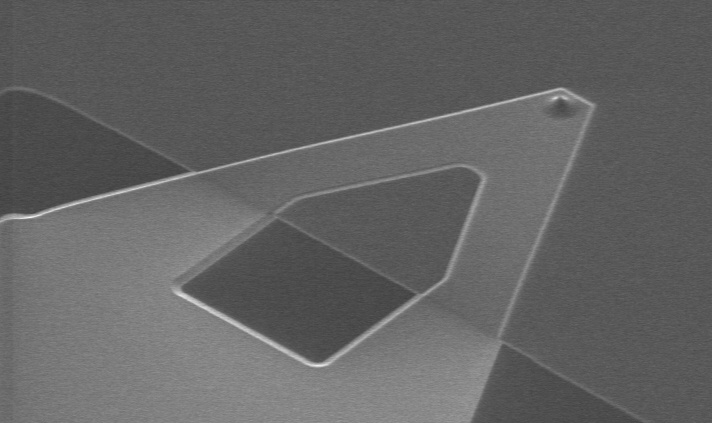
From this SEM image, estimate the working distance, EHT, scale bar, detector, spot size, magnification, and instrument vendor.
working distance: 3.4 mm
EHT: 10 kV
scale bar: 50000 nm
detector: SE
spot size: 3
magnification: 0.5 K X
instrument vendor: FEI